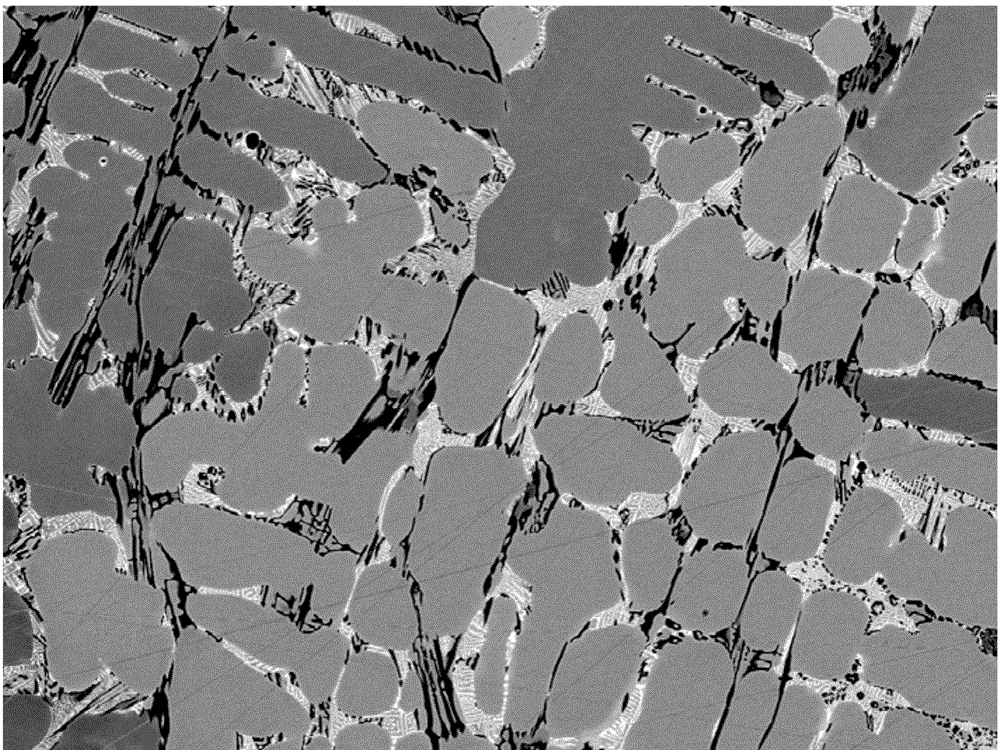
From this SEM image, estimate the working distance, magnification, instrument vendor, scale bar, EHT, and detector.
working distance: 10 mm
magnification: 0.5 K X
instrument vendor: JEOL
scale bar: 10000 nm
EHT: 20 kV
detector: COMPO